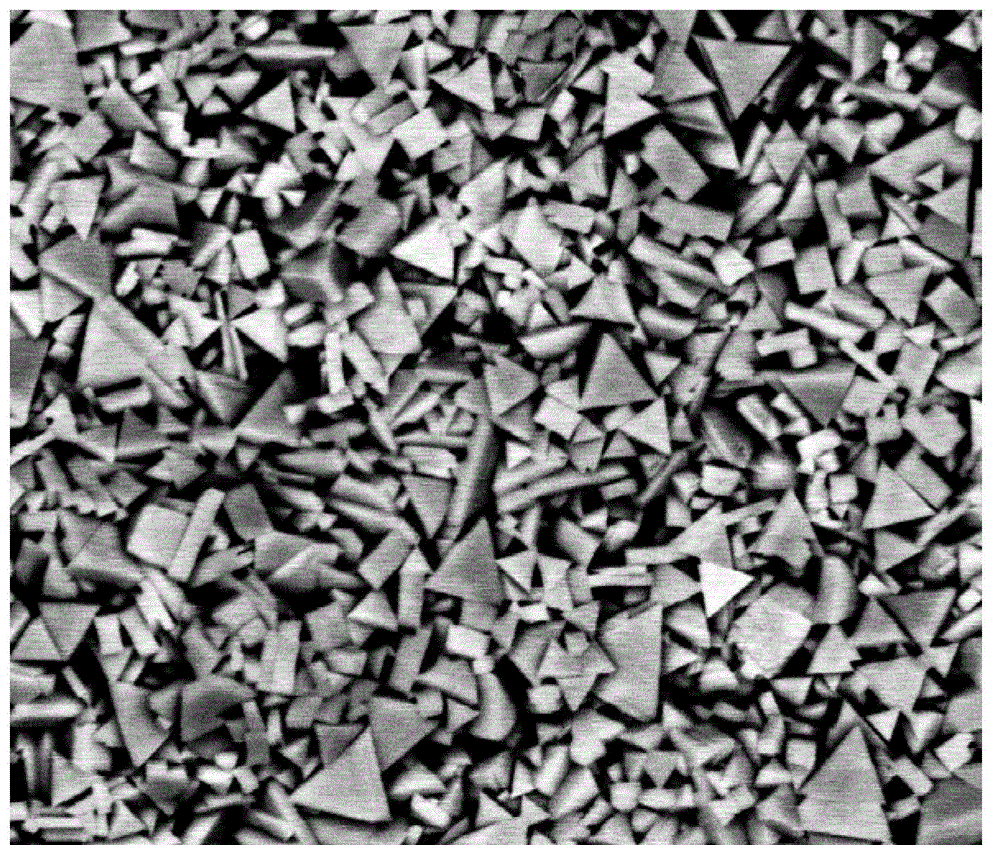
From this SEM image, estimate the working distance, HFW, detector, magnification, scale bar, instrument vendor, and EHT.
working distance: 8.5 mm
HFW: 12 µm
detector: BSED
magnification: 20 K X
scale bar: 3000 nm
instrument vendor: FEI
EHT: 15 kV